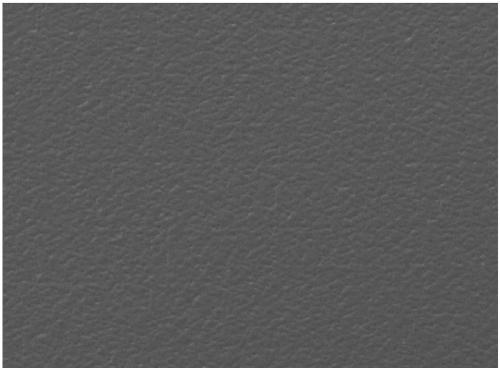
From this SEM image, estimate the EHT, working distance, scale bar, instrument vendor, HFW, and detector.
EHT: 30 kV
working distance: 8.52 mm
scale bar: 200000 nm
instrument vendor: Tescan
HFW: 1040 µm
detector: SE